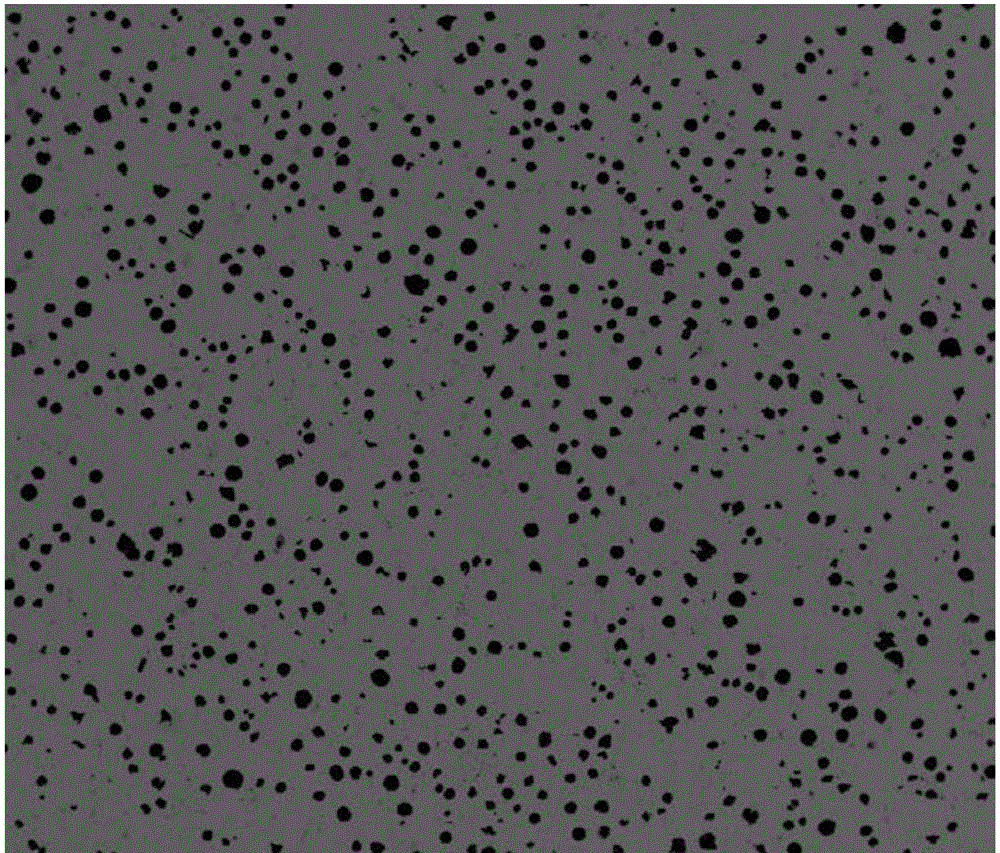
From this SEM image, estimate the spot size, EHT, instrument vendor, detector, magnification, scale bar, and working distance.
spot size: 6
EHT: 20 kV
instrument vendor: FEI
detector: DualBSD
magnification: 0.1 K X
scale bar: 1000000 nm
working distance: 10.4 mm